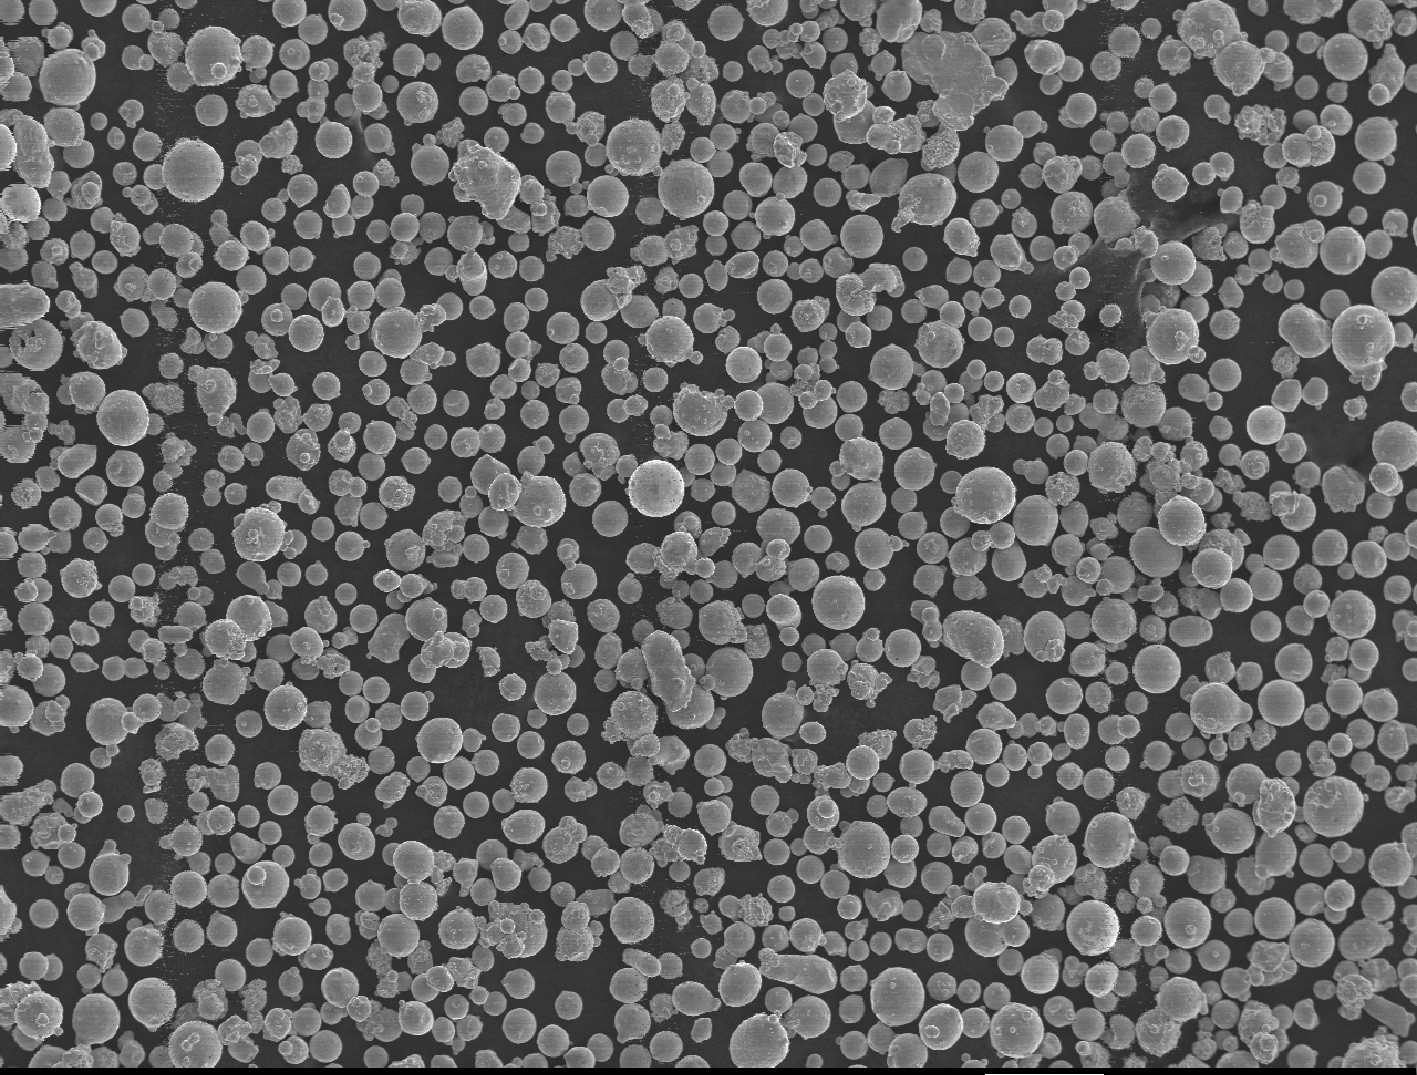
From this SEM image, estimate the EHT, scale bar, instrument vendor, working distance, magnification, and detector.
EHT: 15 kV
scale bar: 100000 nm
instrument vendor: JEOL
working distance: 14.6 mm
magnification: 0.1 K X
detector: SEI